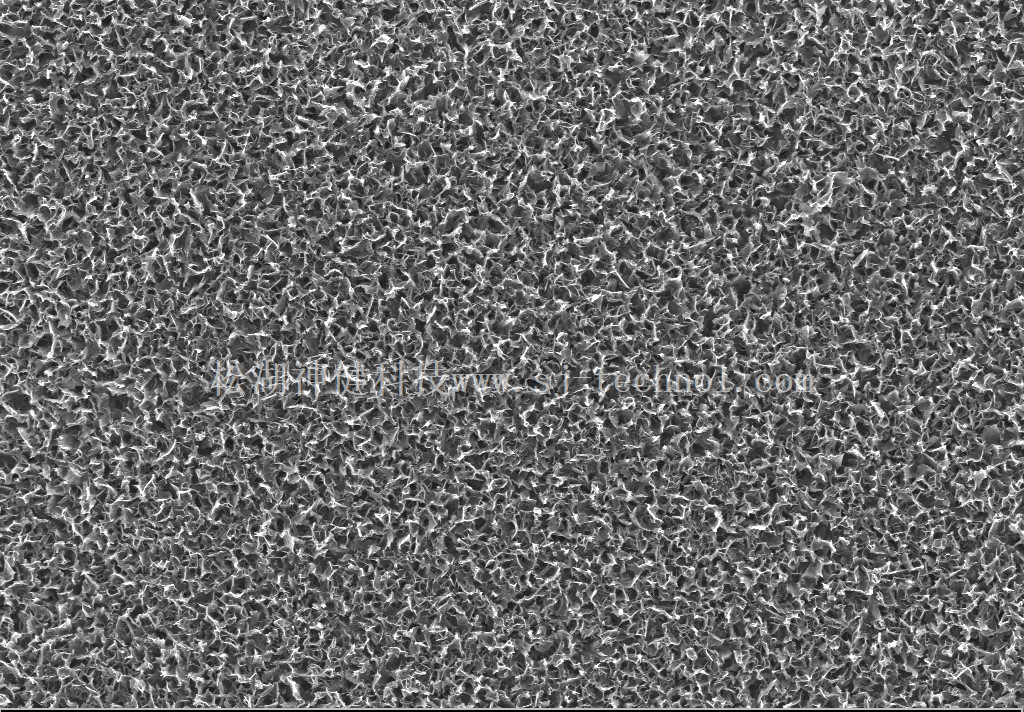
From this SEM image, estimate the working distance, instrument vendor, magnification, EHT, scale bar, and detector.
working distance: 4.8 mm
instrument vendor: Zeiss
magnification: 10 K X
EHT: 3 kV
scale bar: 1000 nm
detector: InLens